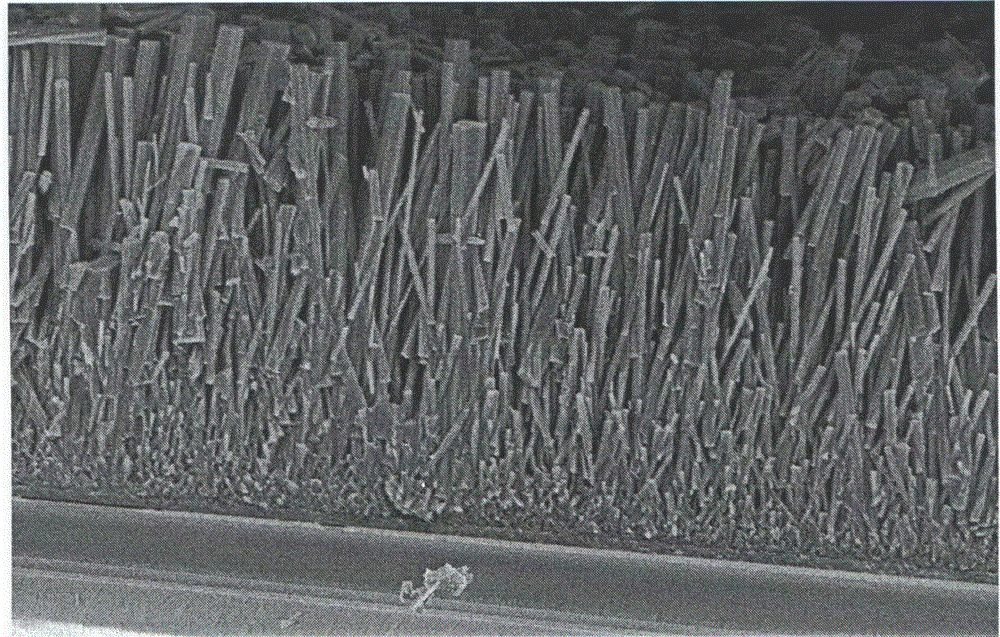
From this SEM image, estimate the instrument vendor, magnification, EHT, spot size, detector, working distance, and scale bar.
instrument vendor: FEI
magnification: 5 K X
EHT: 5 kV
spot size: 3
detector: SE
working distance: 5 mm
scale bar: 5000 nm